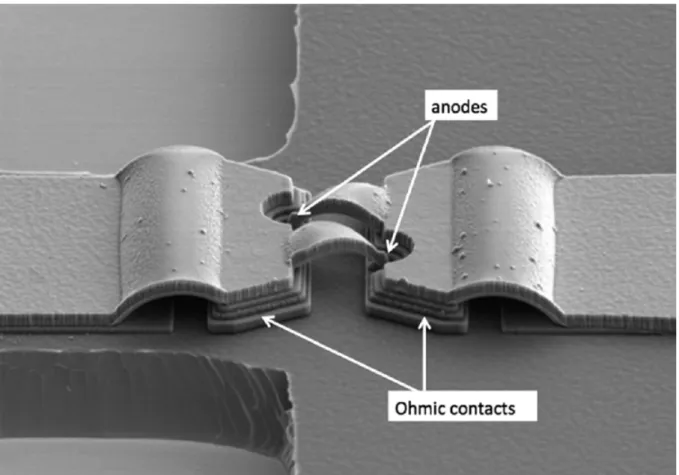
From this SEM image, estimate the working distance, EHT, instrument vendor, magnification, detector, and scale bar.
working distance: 14.7 mm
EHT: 5 kV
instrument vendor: Hitachi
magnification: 3.5 K X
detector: SE(M)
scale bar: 10000 nm